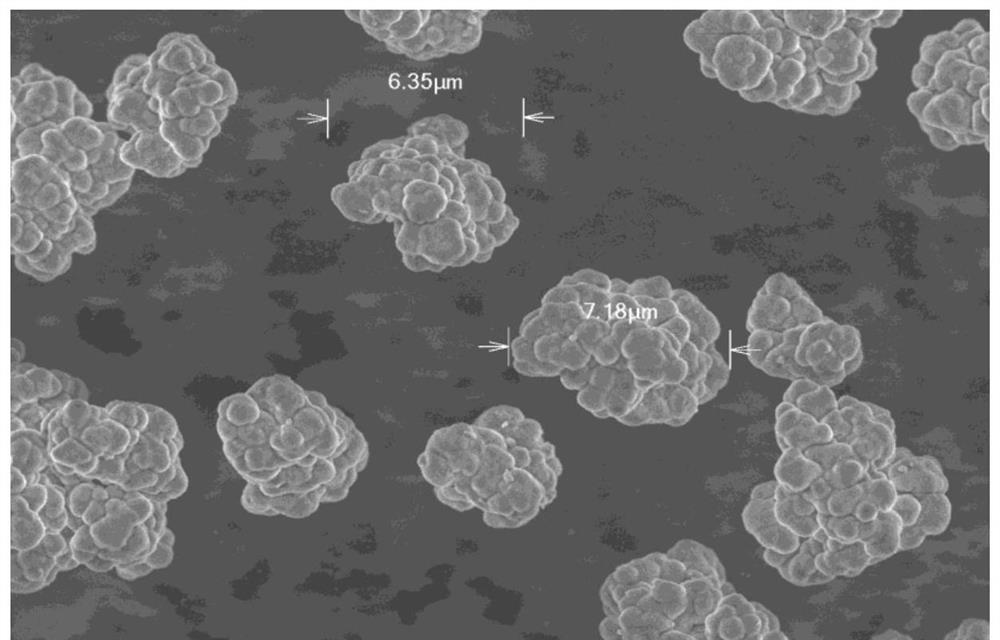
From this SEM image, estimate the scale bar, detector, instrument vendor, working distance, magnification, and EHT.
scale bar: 10000 nm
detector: SE(UL)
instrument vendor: Hitachi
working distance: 4.9 mm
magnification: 4 K X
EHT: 3 kV